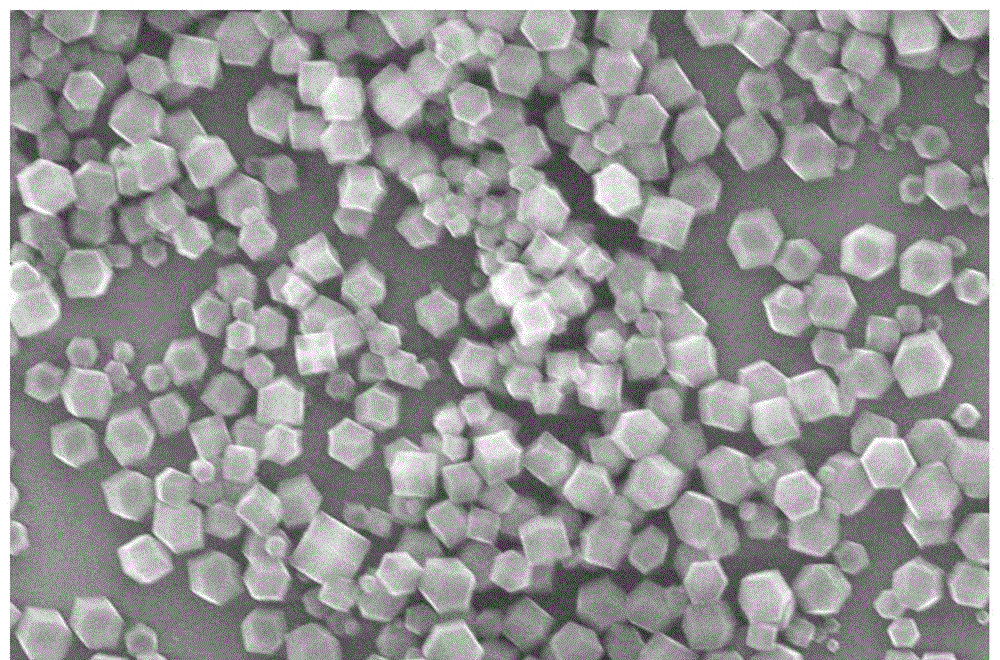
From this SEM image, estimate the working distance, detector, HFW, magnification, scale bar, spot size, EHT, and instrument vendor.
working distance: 12.3 mm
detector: ETD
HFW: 15.9 µm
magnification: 8 K X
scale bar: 5000 nm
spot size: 3.5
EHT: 25 kV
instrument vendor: FEI